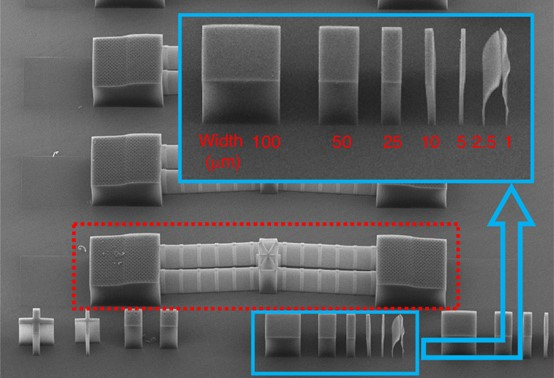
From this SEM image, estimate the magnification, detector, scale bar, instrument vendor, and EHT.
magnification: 0.08 K X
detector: LM(L)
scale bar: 500000 nm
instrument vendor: Hitachi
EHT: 5 kV